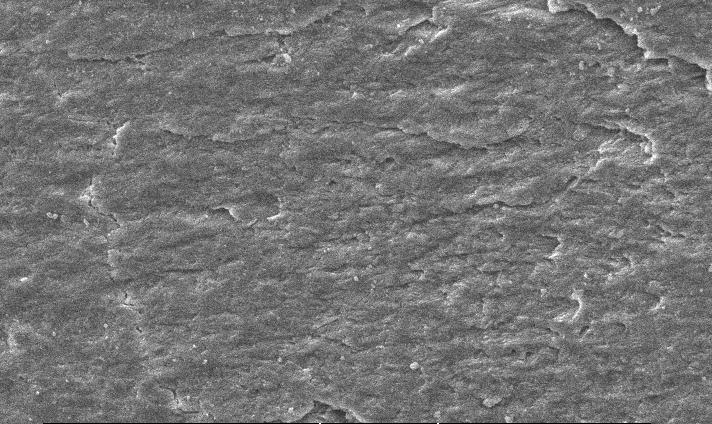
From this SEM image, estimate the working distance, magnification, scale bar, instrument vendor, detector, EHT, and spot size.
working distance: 11 mm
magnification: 0.8 K X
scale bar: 20000 nm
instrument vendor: FEI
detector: SE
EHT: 10 kV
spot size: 4